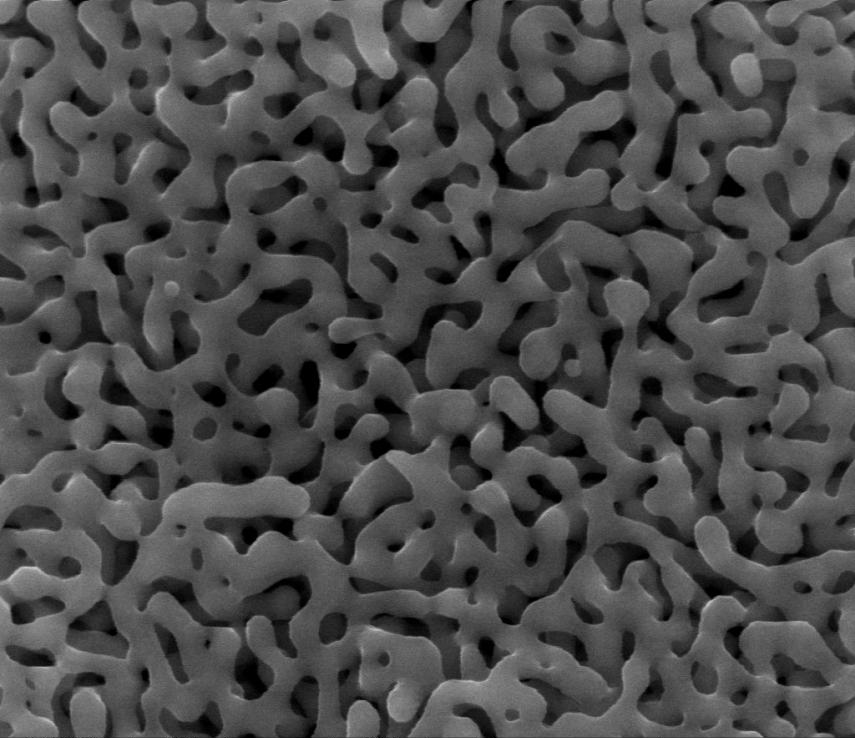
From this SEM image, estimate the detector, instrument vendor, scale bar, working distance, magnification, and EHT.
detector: SE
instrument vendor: FEI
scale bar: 1000 nm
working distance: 10.1 mm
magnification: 100 K X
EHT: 20 kV